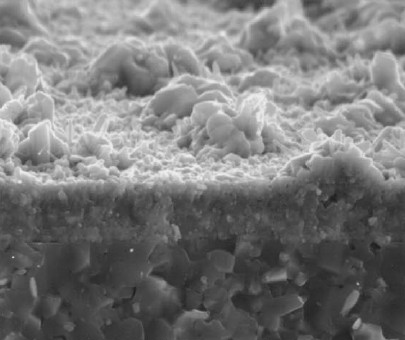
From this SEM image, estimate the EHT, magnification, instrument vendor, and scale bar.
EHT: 15 kV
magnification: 5 K X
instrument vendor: JEOL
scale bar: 5000 nm